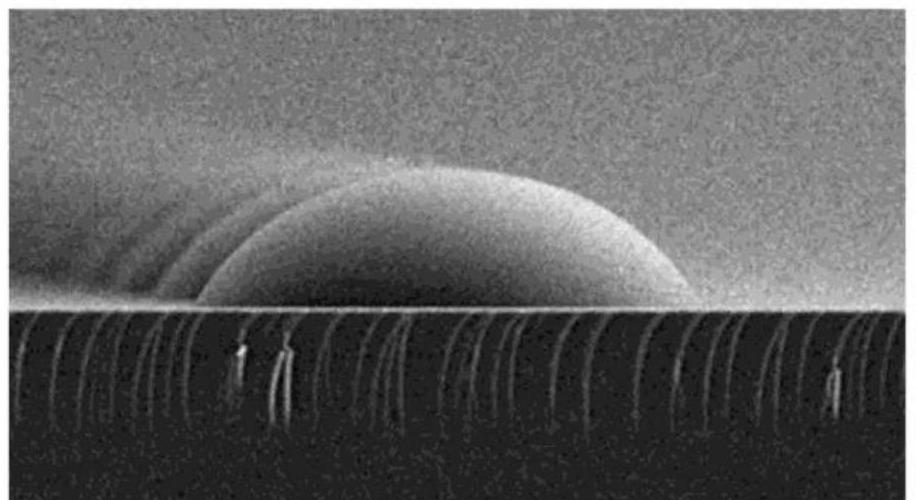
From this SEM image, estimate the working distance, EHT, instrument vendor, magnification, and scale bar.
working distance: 10.6 mm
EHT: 5 kV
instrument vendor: Hitachi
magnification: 3 K X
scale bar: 10000 nm